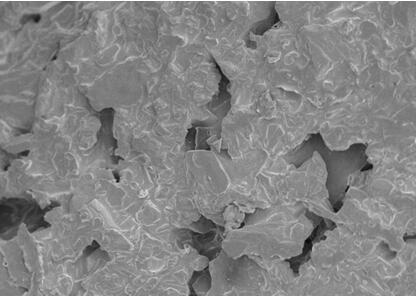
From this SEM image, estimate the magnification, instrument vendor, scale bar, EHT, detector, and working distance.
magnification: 2 K X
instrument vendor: JEOL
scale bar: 10000 nm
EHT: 5 kV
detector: SEI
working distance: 7 mm